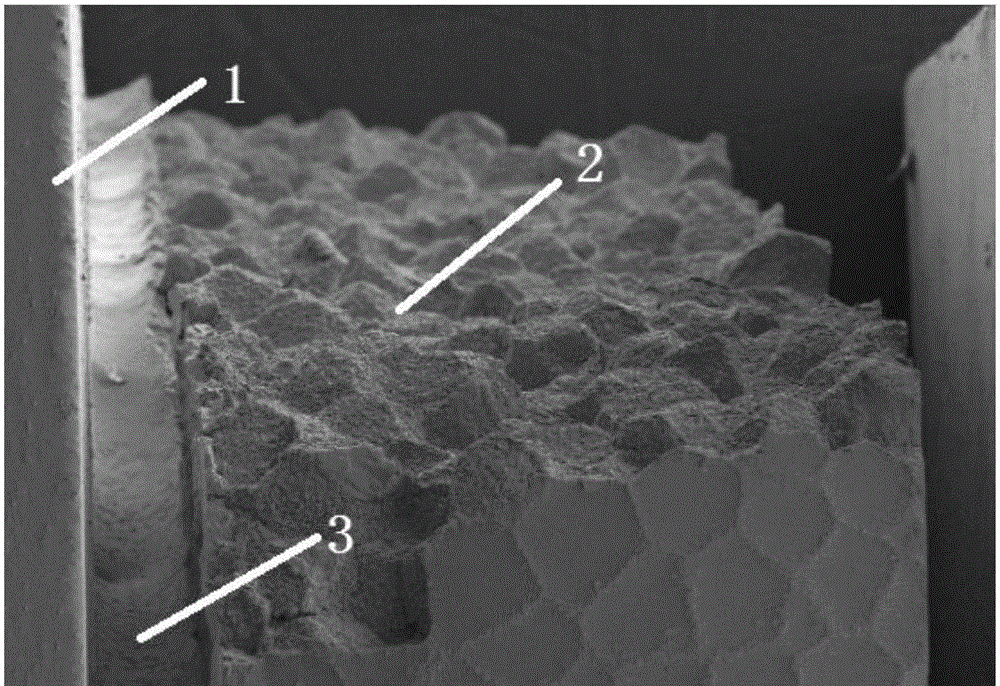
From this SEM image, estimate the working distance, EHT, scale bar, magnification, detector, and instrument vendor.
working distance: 10.5 mm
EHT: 20 kV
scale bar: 100000 nm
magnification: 0.05 K X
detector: SE2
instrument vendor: Zeiss